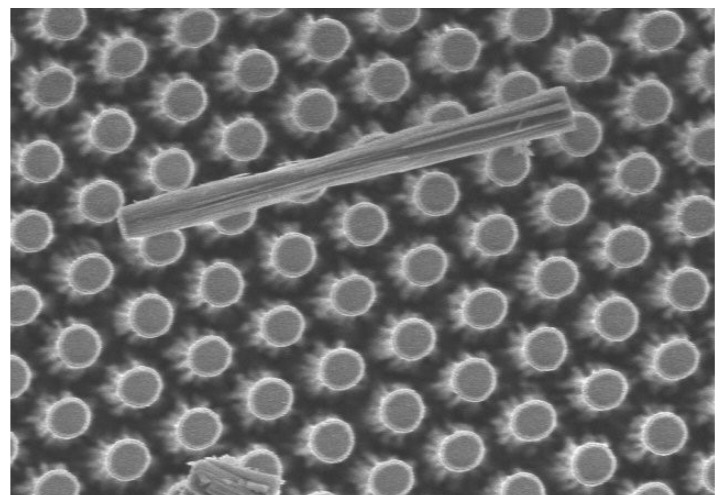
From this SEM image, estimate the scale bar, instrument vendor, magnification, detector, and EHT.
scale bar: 5000 nm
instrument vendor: Hitachi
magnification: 6.01 K X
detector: SE(M)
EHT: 5 kV